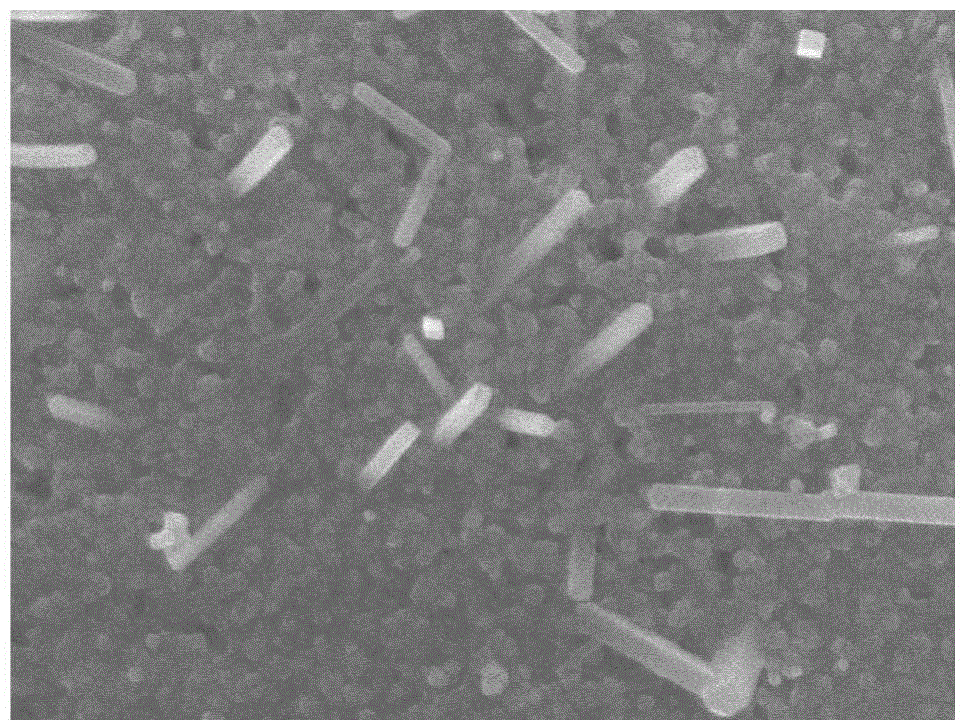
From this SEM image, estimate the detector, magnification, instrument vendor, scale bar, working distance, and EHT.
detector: SEI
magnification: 60 K X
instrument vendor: JEOL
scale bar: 100 nm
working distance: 7.9 mm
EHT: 5 kV